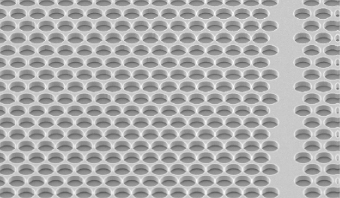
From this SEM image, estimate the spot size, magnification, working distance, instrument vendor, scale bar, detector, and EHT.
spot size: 3.9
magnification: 0.7 K X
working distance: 24.6 mm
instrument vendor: FEI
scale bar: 100000 nm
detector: SE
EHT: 10 kV